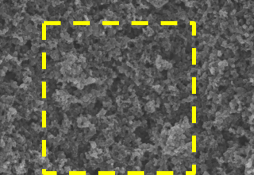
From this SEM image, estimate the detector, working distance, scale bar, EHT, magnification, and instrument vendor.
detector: SE(U)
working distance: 5.1 mm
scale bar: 3000 nm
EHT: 5 kV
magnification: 15 K X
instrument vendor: Hitachi